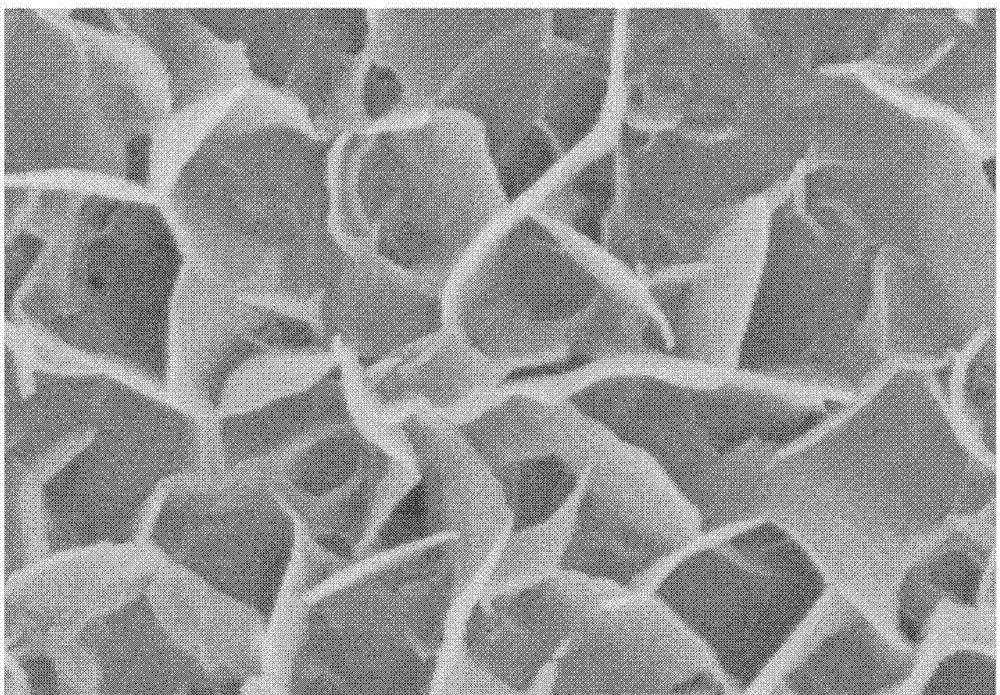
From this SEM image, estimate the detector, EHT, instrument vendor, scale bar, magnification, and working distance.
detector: SE(UL)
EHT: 5 kV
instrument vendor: Hitachi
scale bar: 1000 nm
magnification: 50 K X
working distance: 8.1 mm